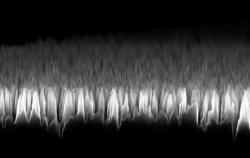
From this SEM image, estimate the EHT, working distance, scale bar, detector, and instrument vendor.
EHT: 10 kV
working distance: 5 mm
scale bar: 1000 nm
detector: InLens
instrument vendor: Zeiss